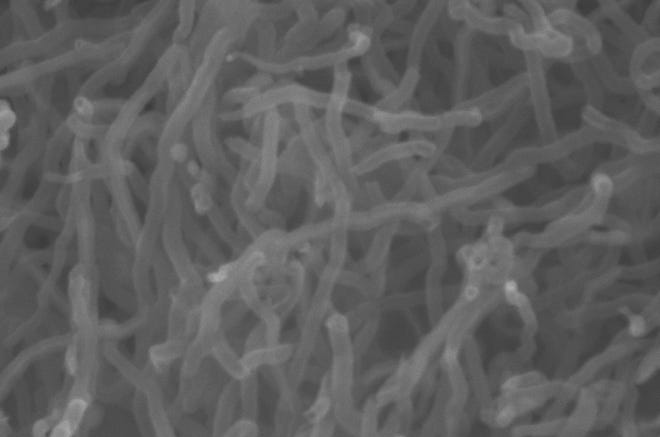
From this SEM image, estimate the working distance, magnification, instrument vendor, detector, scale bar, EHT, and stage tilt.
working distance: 4.1 mm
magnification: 73.73 K X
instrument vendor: Zeiss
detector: InLens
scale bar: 100 nm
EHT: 5 kV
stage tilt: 0°